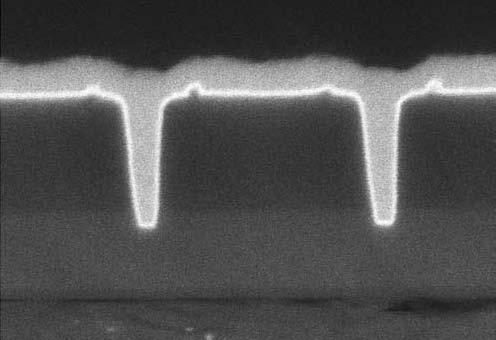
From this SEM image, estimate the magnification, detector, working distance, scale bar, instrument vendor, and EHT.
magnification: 150 K X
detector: SE(U)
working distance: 5.2 mm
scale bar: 300 nm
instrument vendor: Hitachi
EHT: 5 kV